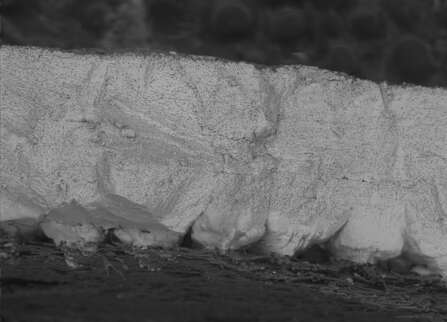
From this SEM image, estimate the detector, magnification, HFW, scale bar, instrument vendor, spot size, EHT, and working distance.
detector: BSE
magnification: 0.25 K X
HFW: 495.9 µm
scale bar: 100000 nm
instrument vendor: FEI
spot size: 3.5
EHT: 10 kV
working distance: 10.6 mm